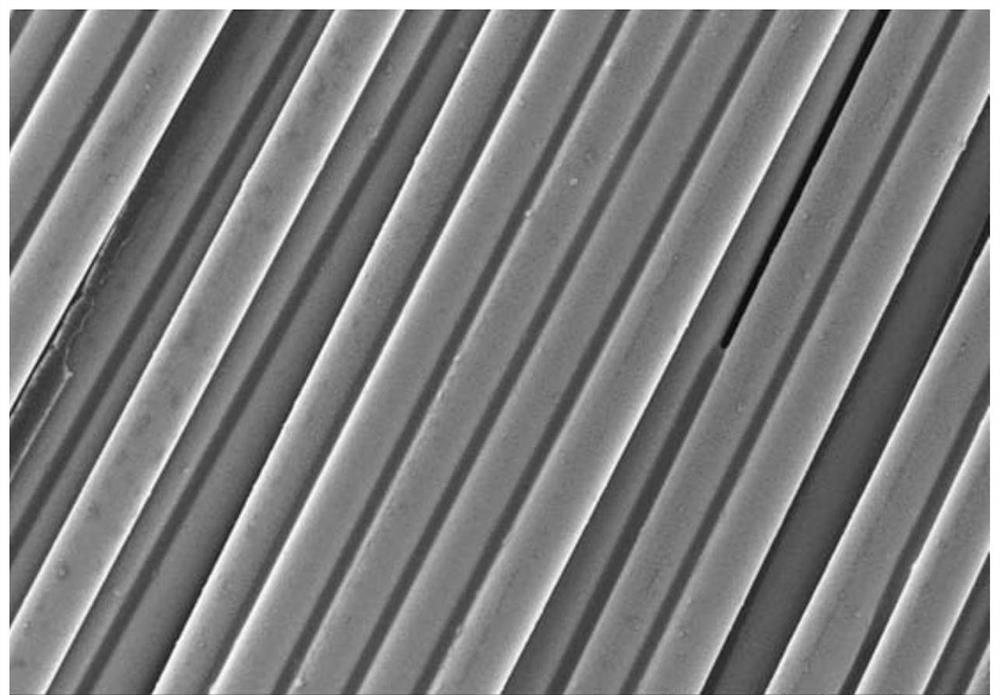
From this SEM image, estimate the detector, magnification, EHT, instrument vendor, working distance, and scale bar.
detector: SE(M)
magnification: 1.2 K X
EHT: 10 kV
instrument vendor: Hitachi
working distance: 7.6 mm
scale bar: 40000 nm